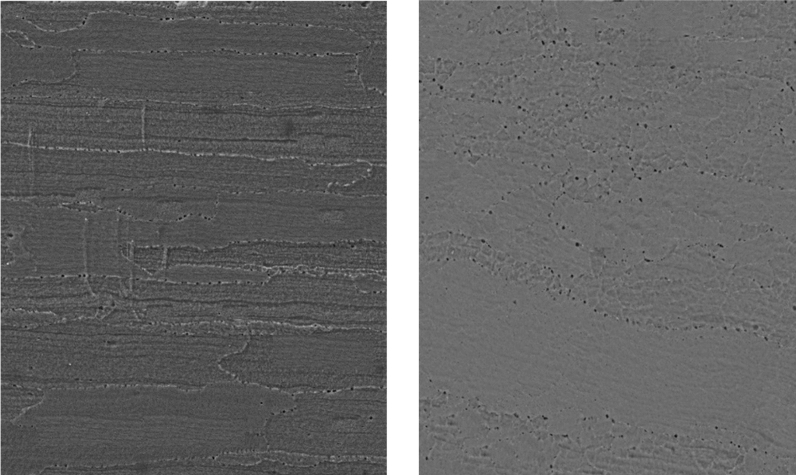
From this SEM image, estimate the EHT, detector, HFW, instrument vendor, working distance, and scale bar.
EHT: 15 kV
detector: SE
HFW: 138 µm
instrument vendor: Tescan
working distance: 14.21 mm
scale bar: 20000 nm